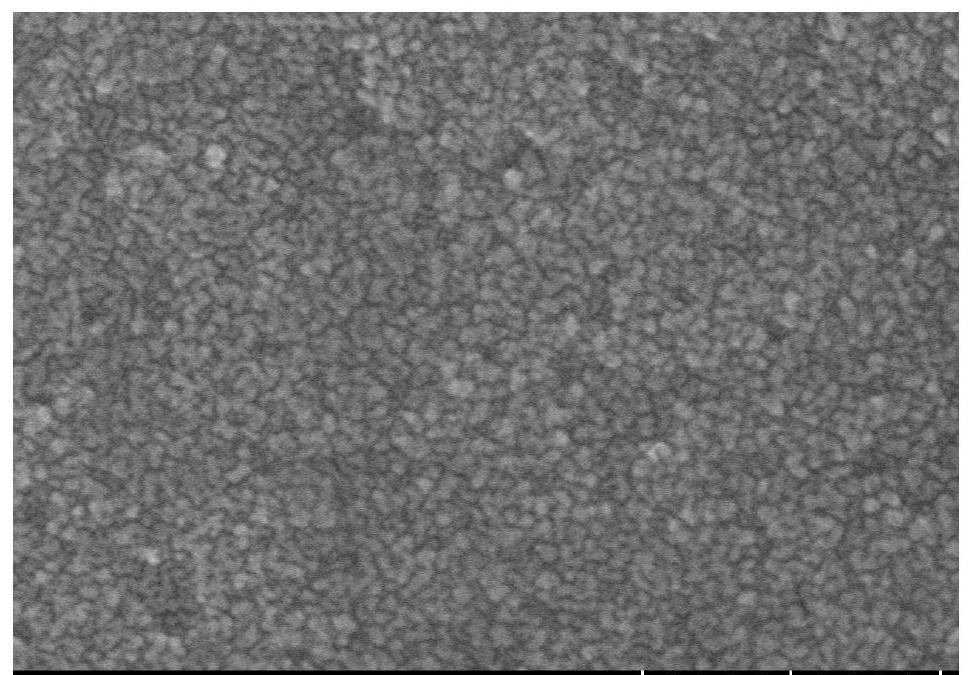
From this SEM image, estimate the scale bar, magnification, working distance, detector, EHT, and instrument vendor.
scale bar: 500 nm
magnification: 80 K X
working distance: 12.9 mm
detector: SE(TUL)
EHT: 10 kV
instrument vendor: Hitachi